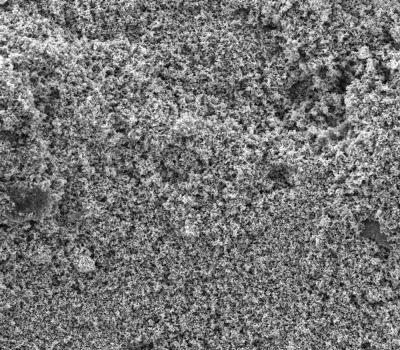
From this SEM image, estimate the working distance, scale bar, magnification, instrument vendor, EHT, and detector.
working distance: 13.56 mm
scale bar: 100000 nm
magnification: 0.5 K X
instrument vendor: Tescan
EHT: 20 kV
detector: SE